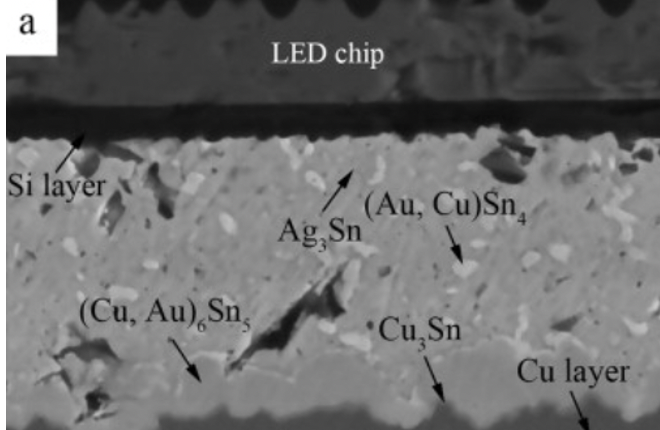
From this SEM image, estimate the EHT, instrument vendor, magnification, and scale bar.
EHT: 15 kV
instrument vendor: JEOL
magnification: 3 K X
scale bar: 5000 nm